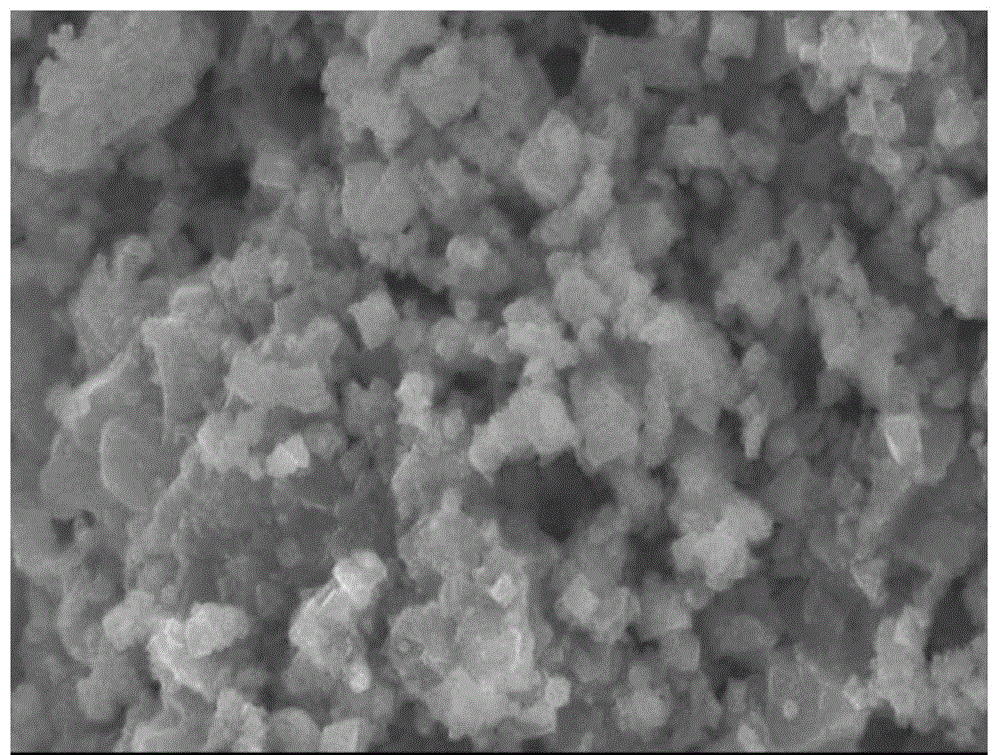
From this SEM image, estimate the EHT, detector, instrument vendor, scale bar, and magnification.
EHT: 30 kV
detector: SEI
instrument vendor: JEOL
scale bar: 5000 nm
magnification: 5 K X